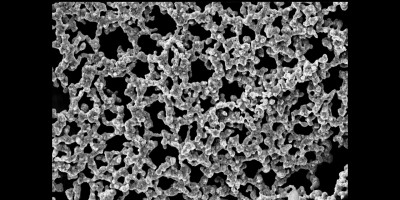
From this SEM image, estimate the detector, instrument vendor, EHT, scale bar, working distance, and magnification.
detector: SE1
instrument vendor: Zeiss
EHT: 20 kV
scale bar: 10000 nm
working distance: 21 mm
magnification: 3.05 K X